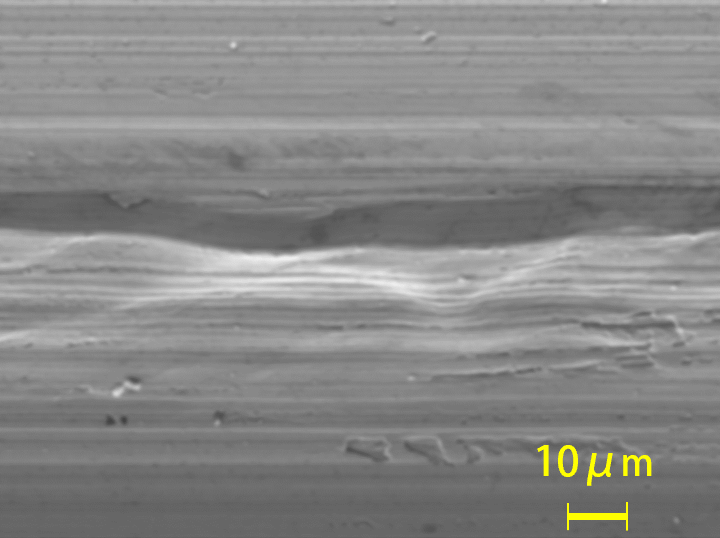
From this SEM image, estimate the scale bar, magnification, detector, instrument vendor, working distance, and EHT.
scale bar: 10000 nm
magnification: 1 K X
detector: SED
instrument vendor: JEOL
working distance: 11.9 mm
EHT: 10 kV